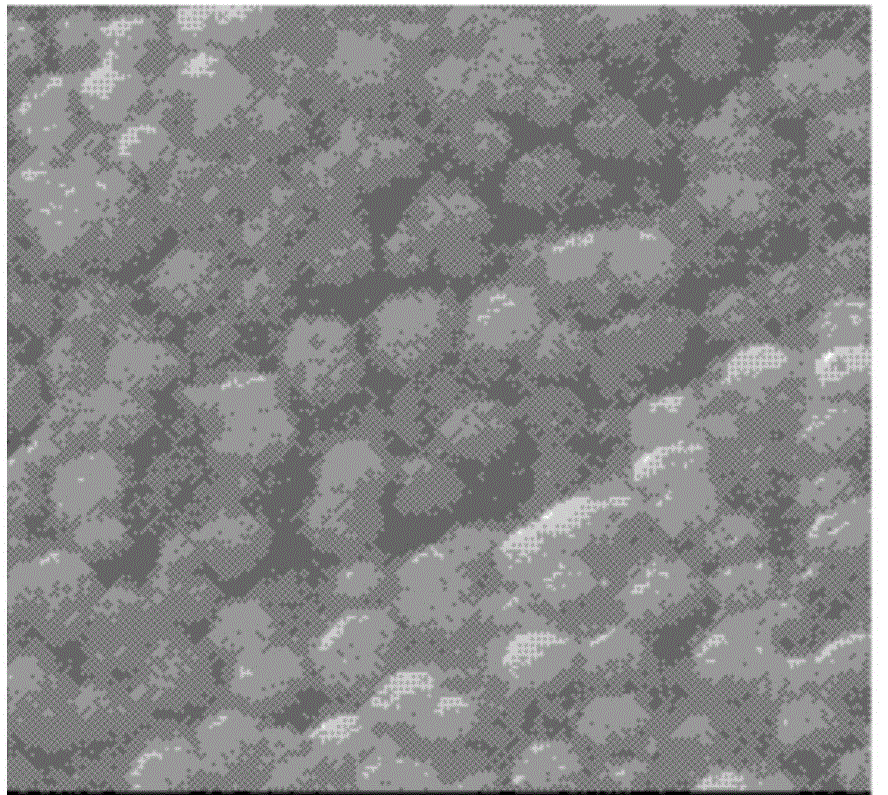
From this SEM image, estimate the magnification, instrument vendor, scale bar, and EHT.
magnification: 3.5 K X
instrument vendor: JEOL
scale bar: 8600 nm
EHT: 15 kV